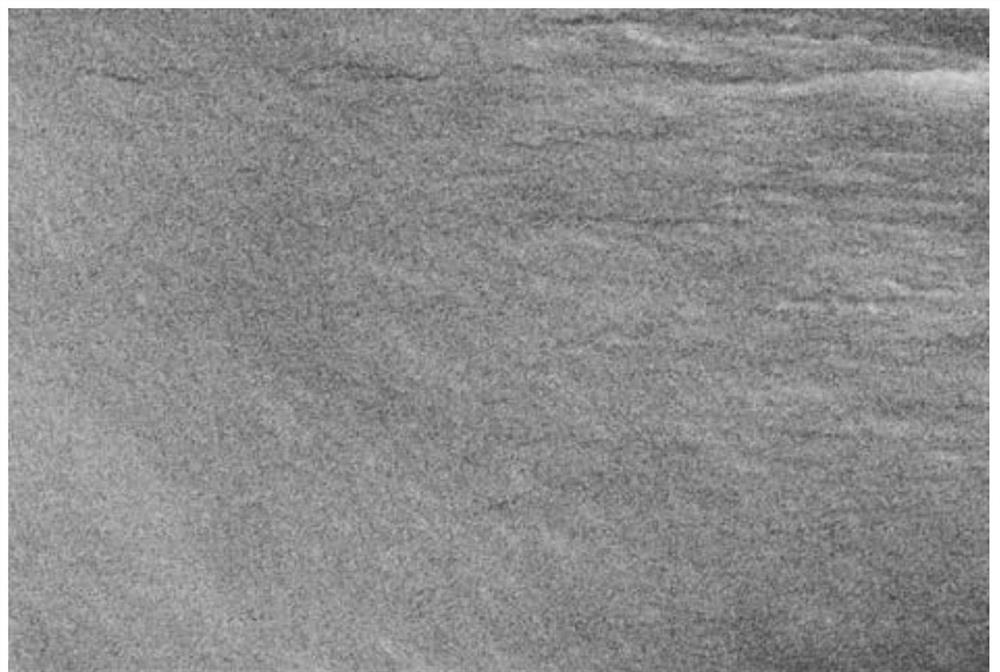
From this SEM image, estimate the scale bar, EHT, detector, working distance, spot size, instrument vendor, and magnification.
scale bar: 1000 nm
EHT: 10 kV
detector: SEI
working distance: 10 mm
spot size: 30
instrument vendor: JEOL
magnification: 10 K X